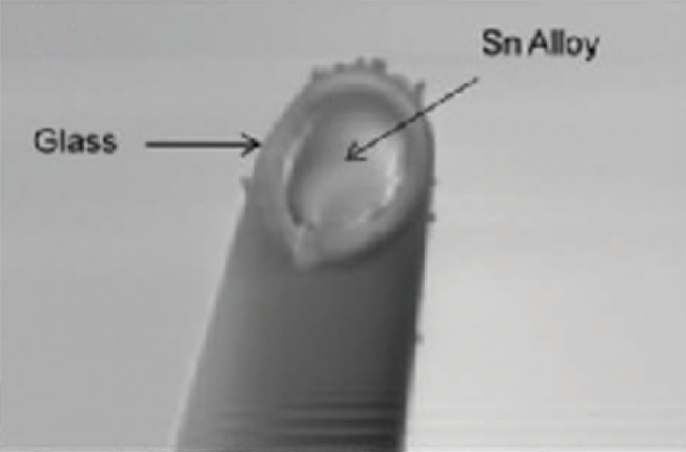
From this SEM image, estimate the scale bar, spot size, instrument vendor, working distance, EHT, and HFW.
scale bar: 5000 nm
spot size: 5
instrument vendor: FEI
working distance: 11.1 mm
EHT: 10 kV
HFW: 20.9 µm